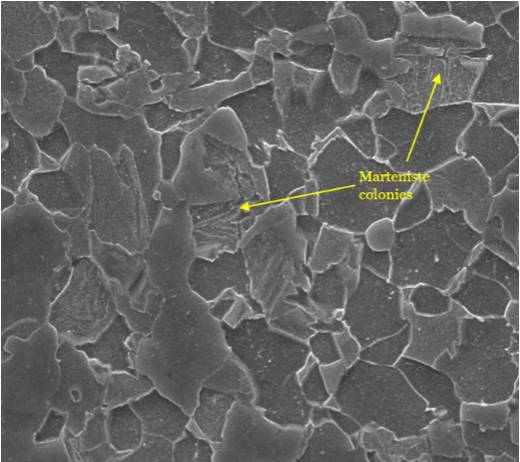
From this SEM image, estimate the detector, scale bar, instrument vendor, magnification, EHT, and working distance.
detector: SE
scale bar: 5000 nm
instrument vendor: FEI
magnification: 20 K X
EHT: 15 kV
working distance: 15.8 mm